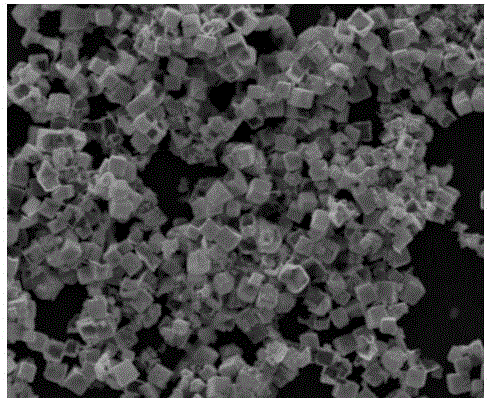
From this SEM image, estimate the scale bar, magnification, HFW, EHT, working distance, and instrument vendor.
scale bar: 10000 nm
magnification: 12 K X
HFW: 24.9 µm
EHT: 10 kV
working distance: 11.3 mm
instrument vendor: FEI